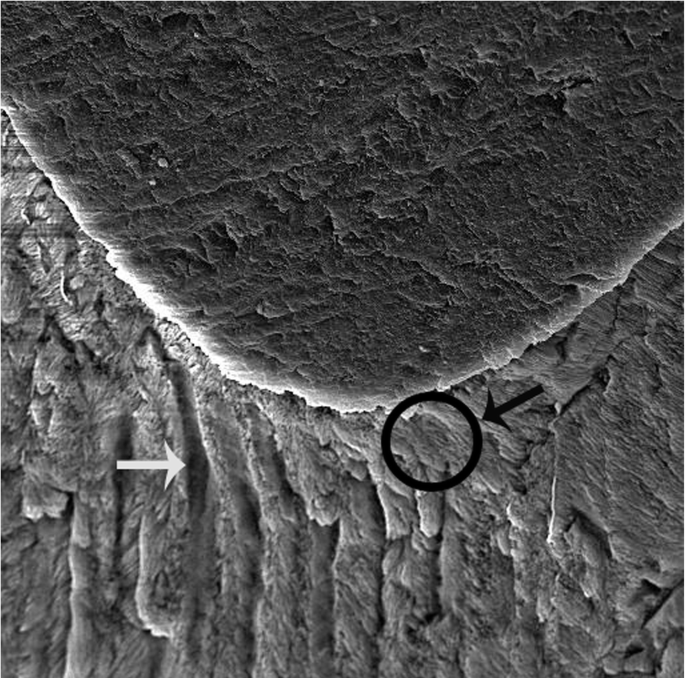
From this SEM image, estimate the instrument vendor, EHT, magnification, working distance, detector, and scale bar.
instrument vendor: Tescan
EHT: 15 kV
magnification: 1.5 K X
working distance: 8.326 mm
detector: SE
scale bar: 20000 nm